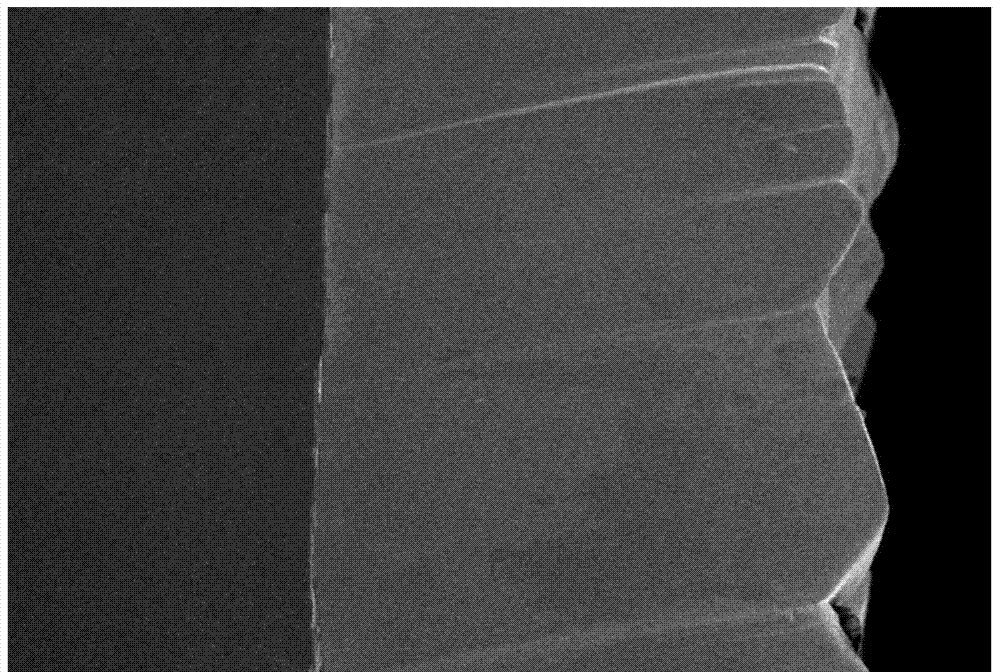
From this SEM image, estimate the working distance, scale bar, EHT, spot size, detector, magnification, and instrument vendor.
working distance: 11 mm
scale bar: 50000 nm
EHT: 10 kV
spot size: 40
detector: SEI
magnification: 0.3 K X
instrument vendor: JEOL